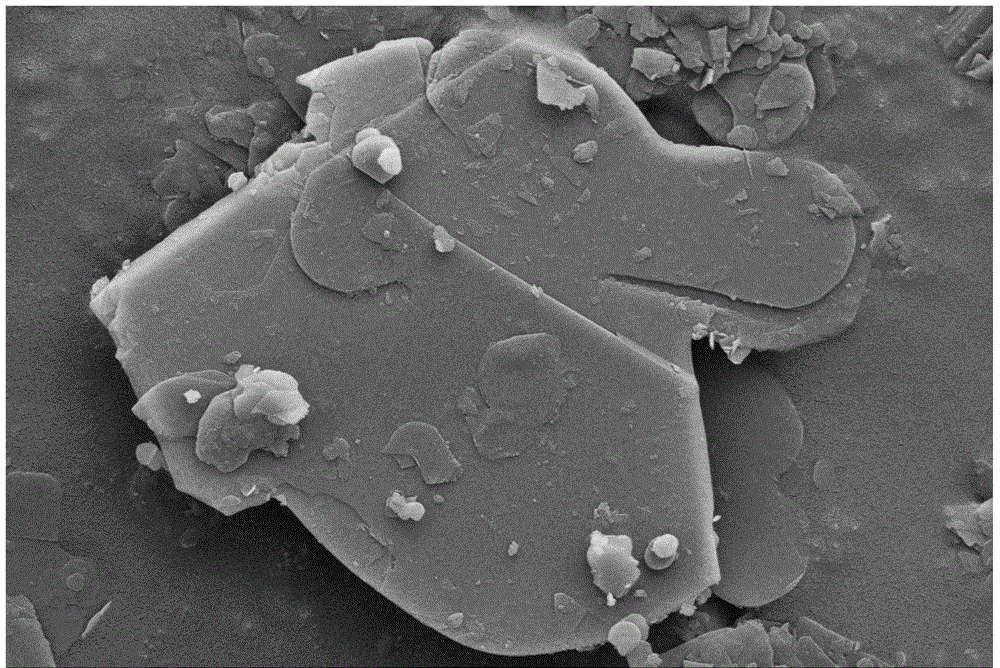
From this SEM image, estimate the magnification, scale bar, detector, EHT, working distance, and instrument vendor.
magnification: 8 K X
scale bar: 2000 nm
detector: SE2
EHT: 5 kV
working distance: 7.4 mm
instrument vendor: Zeiss